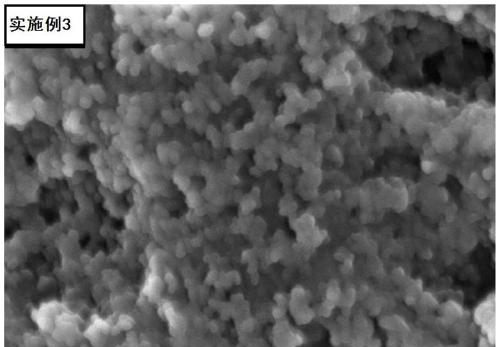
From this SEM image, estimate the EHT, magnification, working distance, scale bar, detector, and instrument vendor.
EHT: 5 kV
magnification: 70 K X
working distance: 3.7 mm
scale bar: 100 nm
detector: InLens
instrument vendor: Zeiss